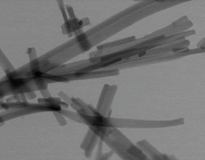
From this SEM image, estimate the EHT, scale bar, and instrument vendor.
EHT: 30 kV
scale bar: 3000 nm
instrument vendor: FEI